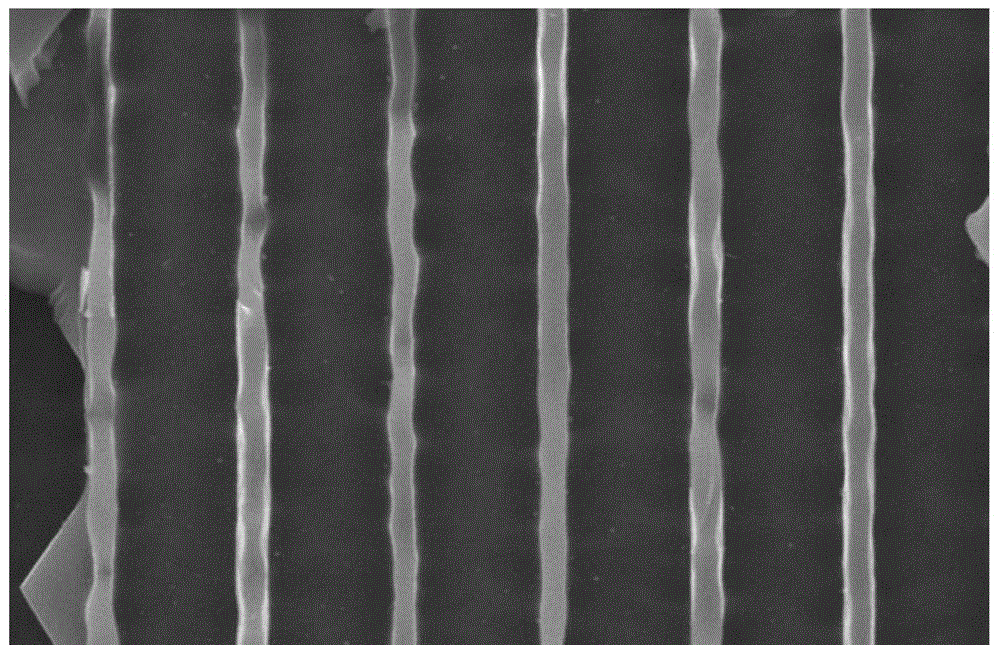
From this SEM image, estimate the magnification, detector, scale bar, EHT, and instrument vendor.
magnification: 5 K X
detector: SEI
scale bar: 5000 nm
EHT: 10 kV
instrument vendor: JEOL